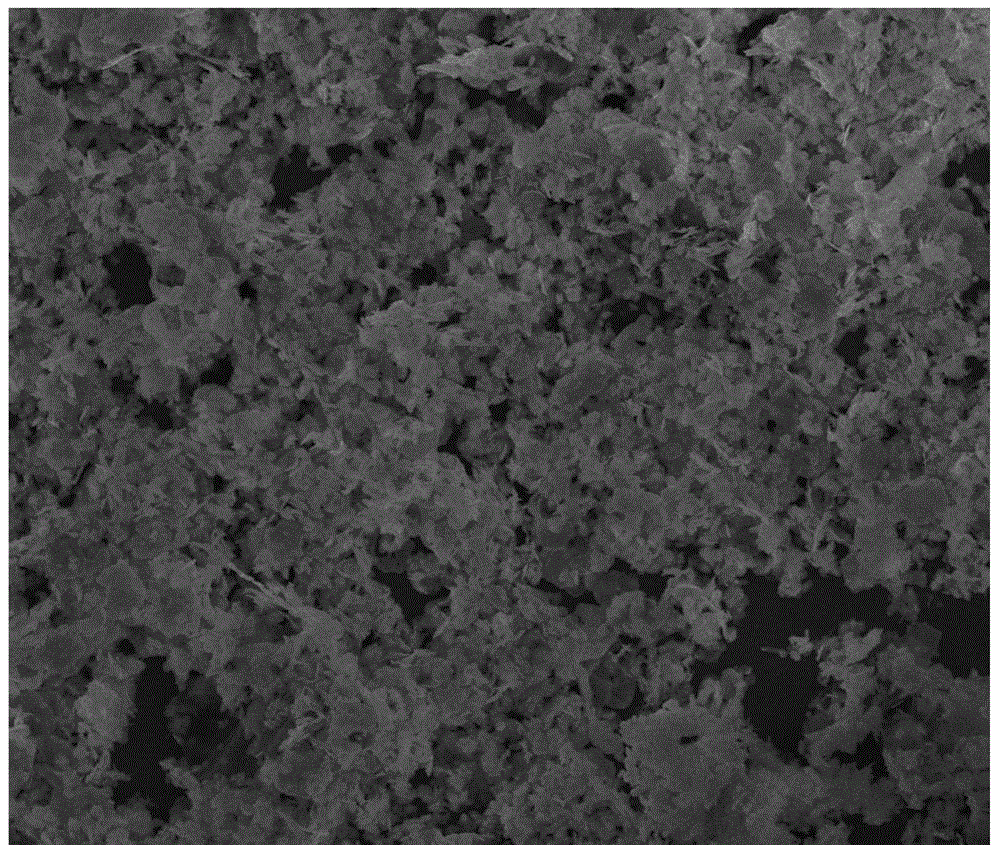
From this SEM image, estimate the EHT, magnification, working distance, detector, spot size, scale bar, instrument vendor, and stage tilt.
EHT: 15 kV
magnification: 5 K X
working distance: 5.5 mm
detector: SE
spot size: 3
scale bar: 10000 nm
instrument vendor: FEI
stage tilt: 0°